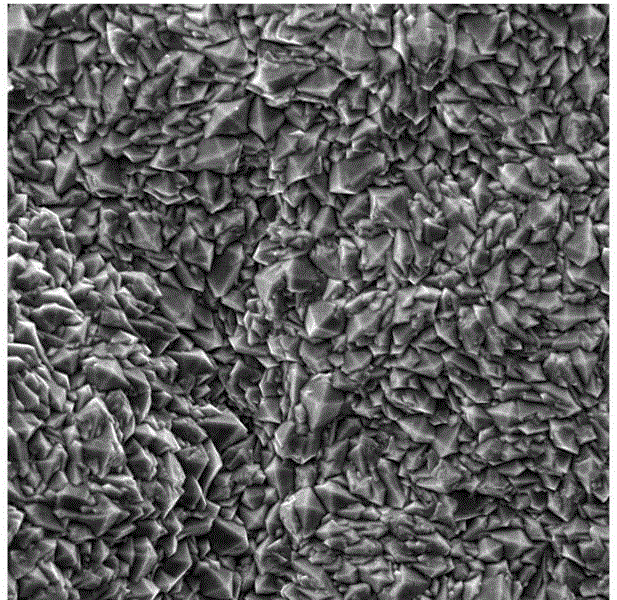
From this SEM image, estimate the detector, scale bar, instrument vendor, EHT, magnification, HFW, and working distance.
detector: SE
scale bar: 20000 nm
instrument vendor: Tescan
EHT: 30 kV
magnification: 2 K X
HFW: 104 µm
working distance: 13 mm